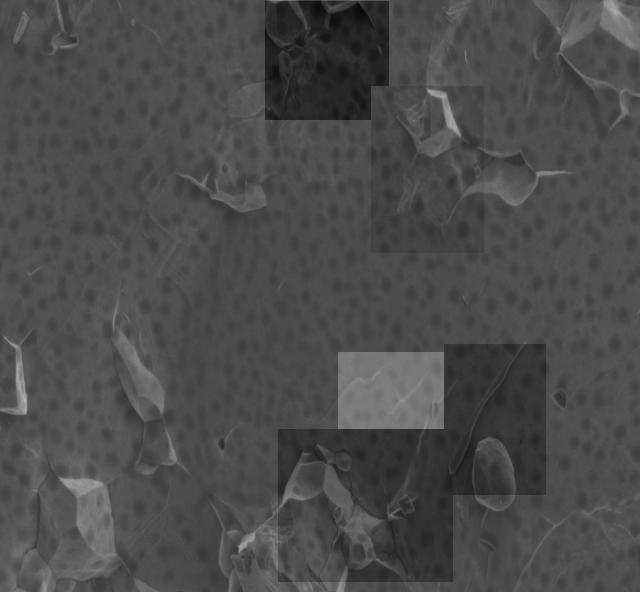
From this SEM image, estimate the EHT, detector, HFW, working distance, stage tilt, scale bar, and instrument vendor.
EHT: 2 kV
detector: ETD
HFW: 20.7 µm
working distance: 4.5 mm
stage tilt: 0°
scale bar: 5000 nm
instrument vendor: FEI